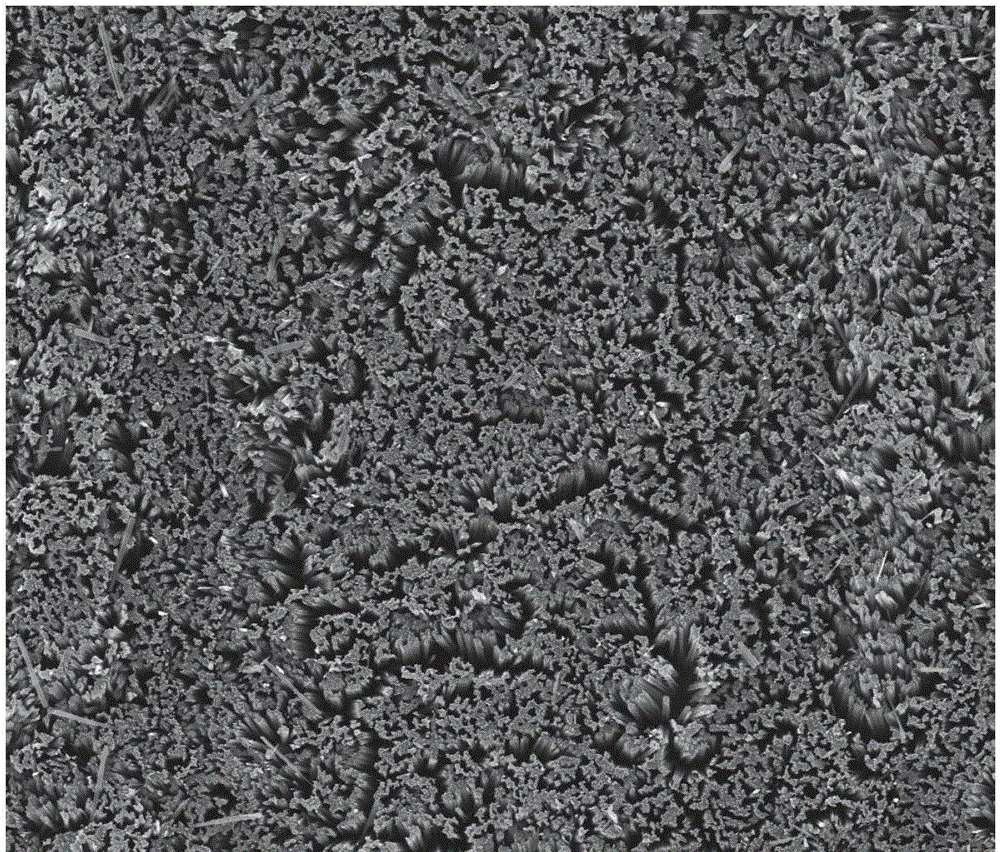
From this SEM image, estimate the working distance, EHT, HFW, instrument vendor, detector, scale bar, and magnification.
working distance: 15.7 mm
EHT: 30 kV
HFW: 128 µm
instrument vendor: FEI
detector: ETD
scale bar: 50000 nm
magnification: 2 K X